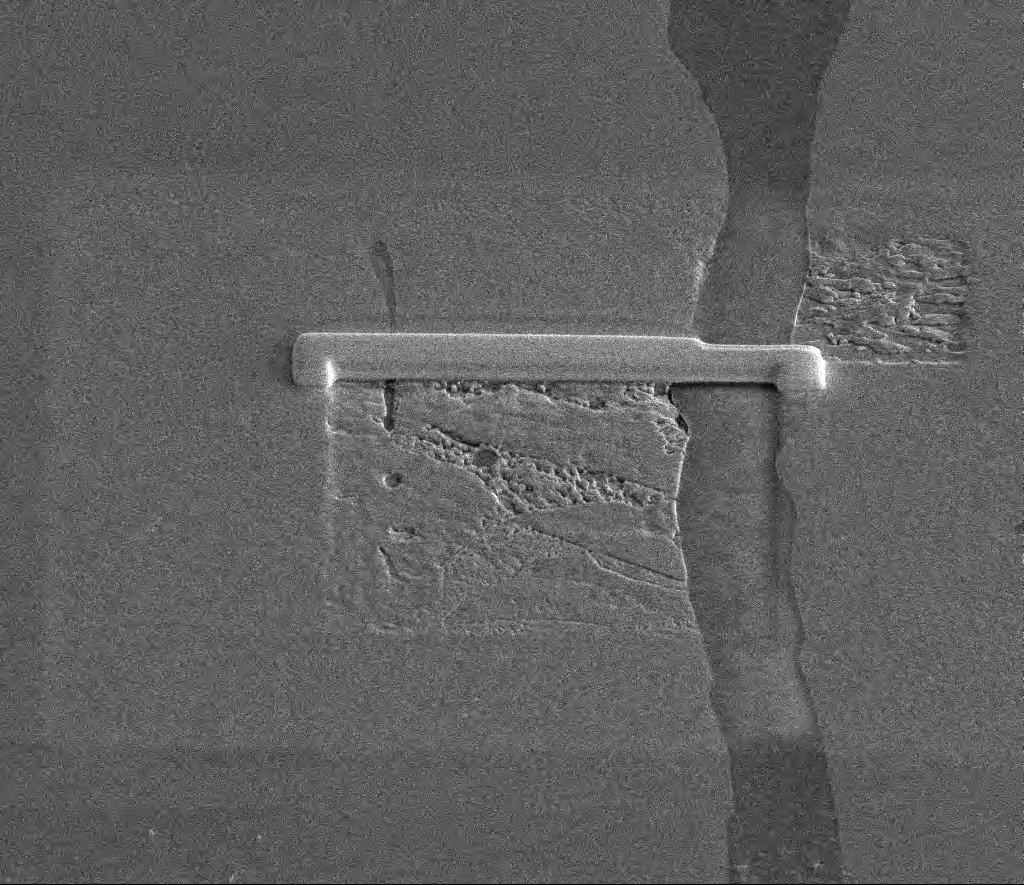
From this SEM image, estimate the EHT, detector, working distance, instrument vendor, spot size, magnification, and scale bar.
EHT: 10 kV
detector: ETD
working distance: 10 mm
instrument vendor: FEI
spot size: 4.5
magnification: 2.5 K X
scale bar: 20000 nm